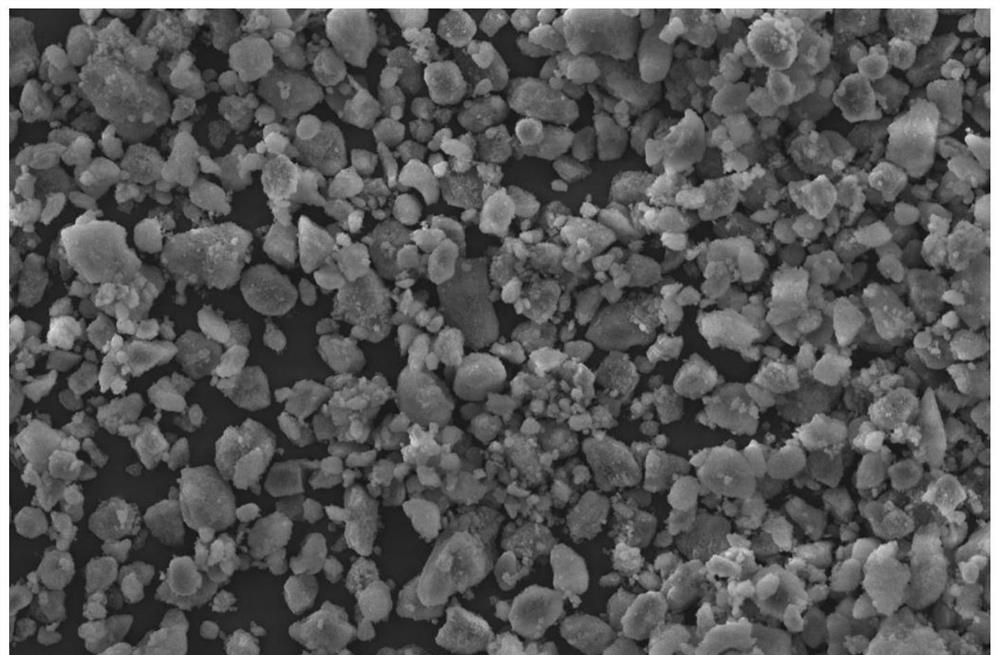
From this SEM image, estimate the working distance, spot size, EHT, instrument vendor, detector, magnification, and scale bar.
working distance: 9.7 mm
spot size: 3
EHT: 10 kV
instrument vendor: FEI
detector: ETD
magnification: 4 K X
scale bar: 30000 nm